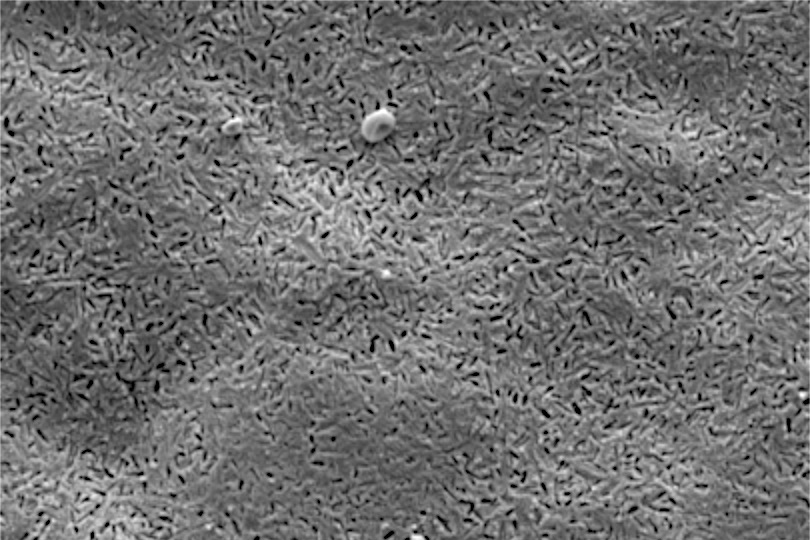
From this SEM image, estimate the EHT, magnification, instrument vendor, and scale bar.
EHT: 10 kV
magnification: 3 K X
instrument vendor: JEOL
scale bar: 5000 nm